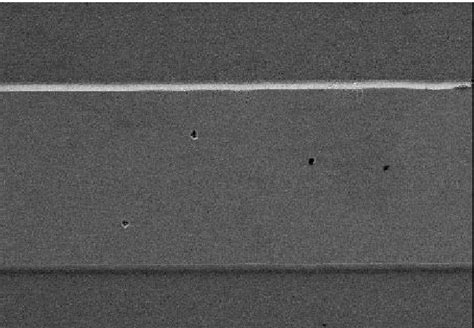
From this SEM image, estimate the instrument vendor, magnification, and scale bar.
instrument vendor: Zeiss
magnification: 5 K X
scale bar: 1000 nm